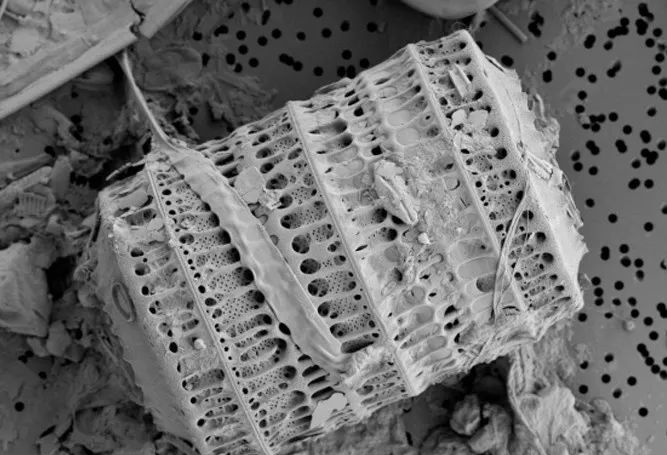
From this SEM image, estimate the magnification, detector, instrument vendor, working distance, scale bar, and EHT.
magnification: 1.8 K X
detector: SE(L)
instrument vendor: Hitachi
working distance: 14.7 mm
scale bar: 30000 nm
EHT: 1.5 kV